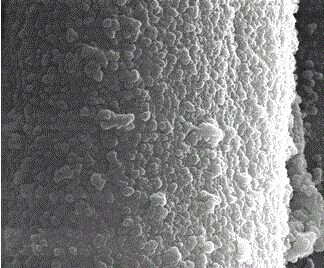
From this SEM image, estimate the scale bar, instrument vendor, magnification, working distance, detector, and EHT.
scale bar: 5000 nm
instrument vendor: FEI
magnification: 25 K X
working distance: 8.8 mm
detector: SE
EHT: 30 kV